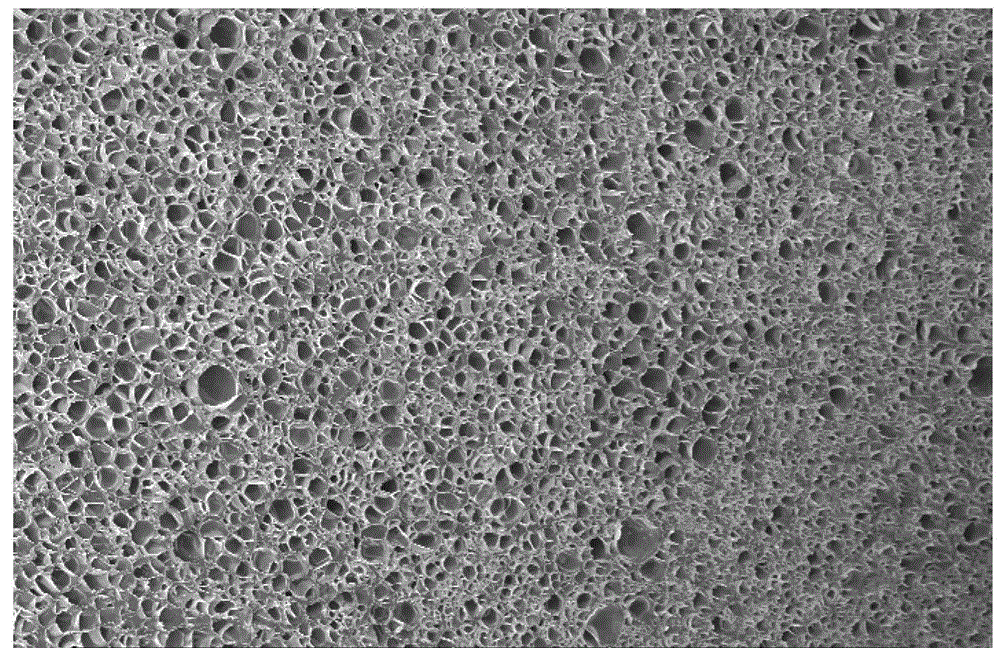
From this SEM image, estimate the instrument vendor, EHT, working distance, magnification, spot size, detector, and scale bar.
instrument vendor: FEI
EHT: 5 kV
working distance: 21.6 mm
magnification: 0.1 K X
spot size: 3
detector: SE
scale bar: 200000 nm